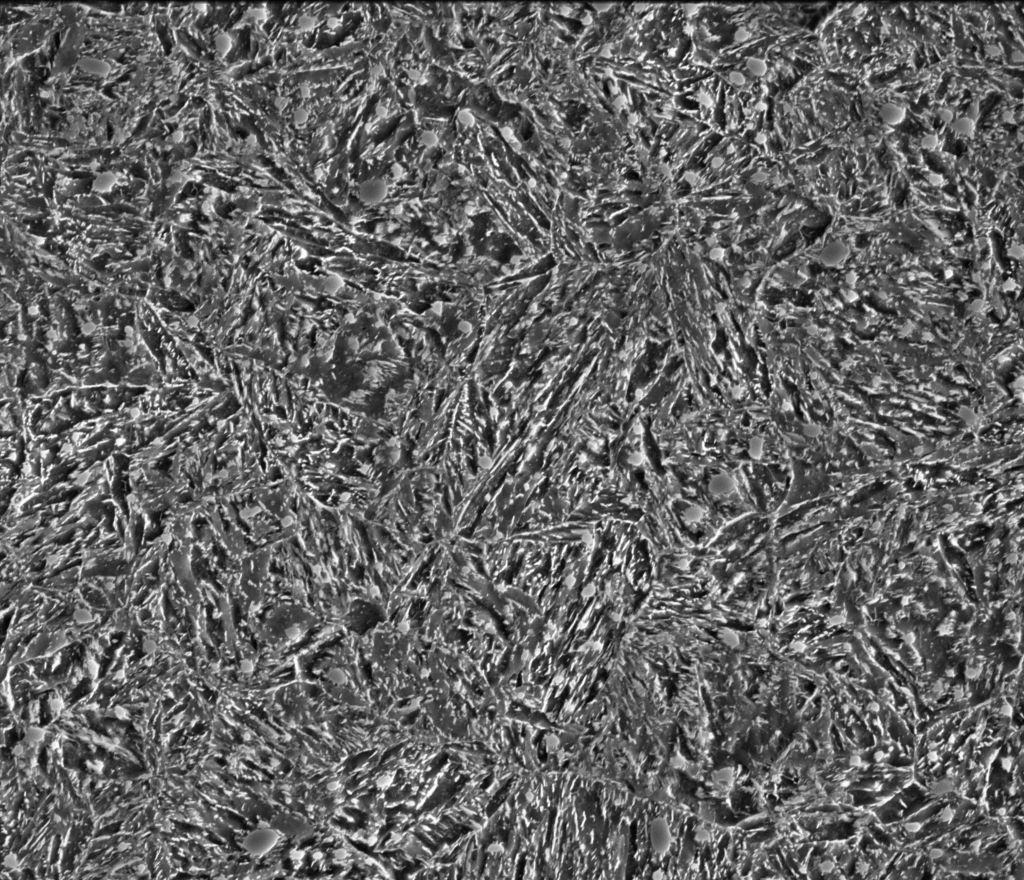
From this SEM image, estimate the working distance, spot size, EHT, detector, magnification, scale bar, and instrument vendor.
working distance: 10 mm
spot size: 5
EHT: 15 kV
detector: ETD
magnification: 10 K X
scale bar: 10000 nm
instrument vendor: FEI